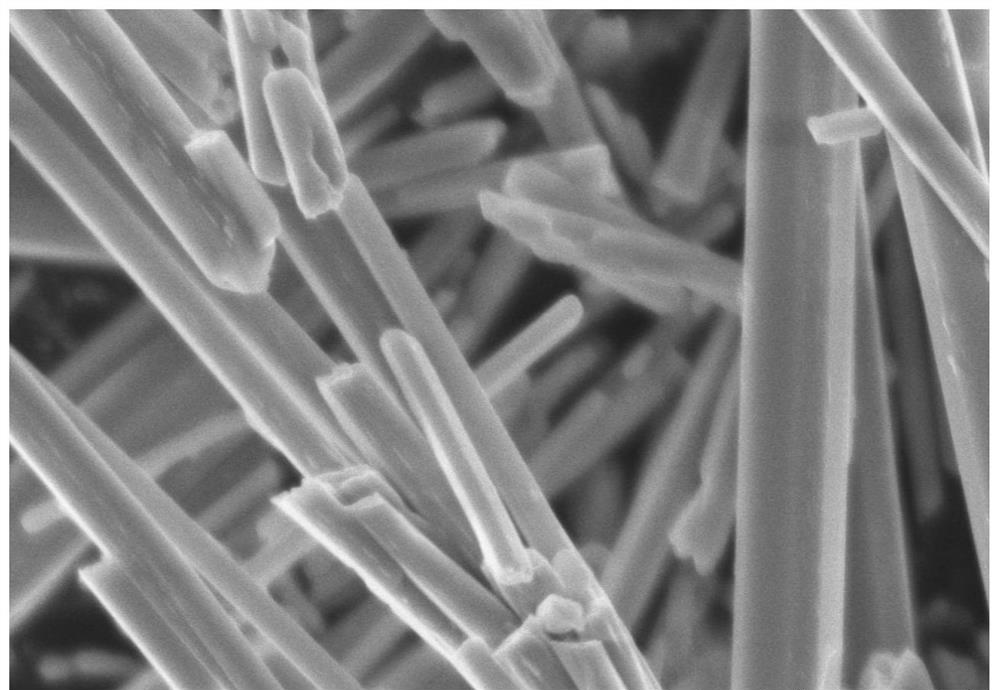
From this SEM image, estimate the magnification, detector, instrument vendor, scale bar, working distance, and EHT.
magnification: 22 K X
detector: SE(U)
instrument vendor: Hitachi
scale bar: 2000 nm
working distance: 8.2 mm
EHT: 5 kV